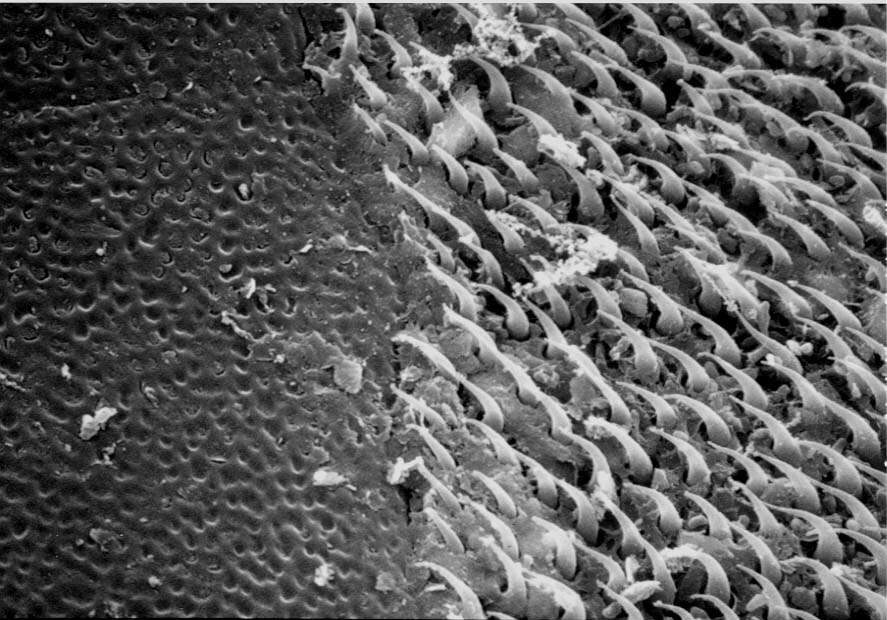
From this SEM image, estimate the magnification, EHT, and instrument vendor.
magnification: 5 K X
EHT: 20 kV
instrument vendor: JEOL